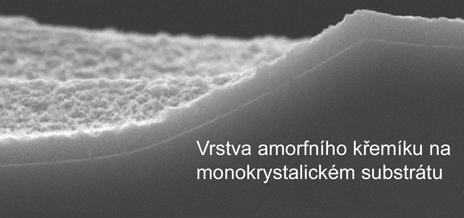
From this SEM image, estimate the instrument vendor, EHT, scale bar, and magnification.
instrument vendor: JEOL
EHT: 10 kV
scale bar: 1000 nm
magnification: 20 K X